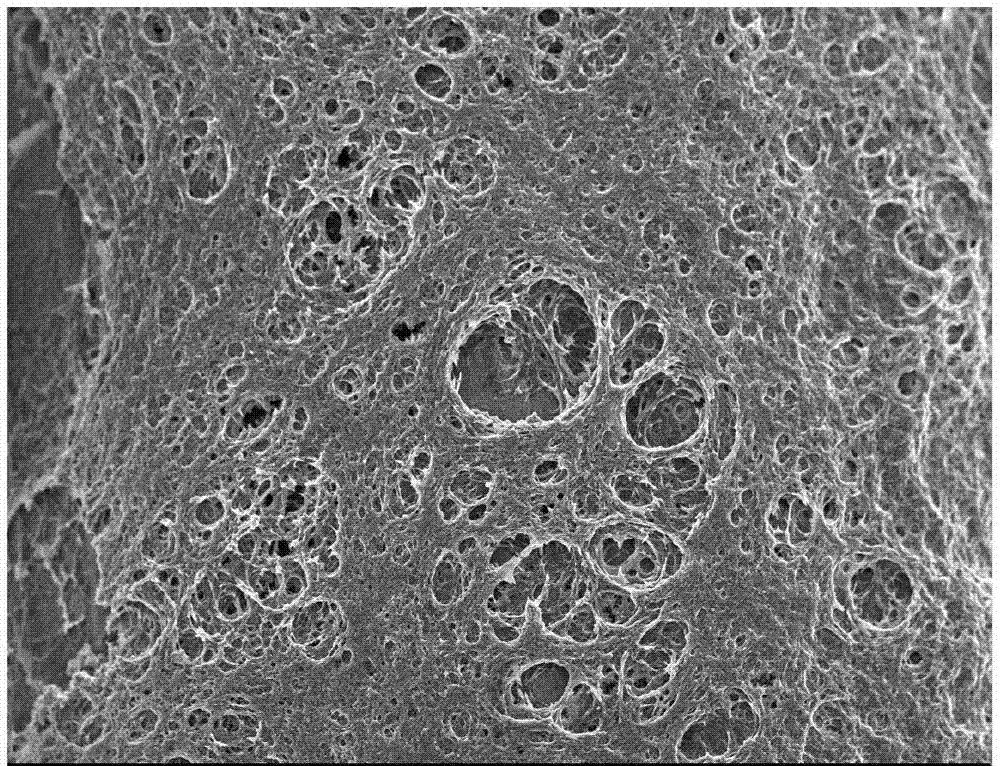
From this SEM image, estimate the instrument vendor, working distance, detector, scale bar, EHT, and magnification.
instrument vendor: JEOL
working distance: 5.6 mm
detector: SEI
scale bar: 10000 nm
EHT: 3 kV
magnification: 2.5 K X